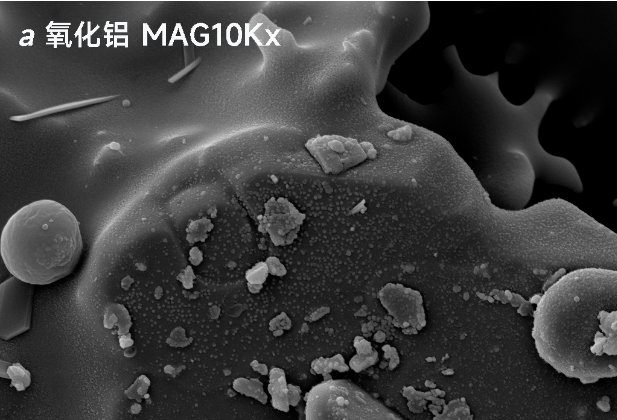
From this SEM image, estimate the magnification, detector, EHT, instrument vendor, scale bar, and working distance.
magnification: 10 K X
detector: SE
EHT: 10 kV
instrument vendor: other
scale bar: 3000 nm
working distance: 8.216 mm